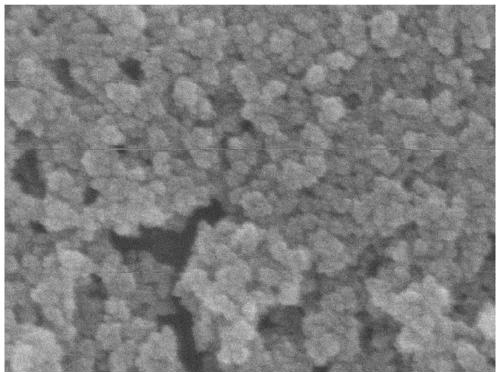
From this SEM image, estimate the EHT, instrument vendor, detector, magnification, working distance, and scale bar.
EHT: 5 kV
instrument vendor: JEOL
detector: SEI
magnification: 160 K X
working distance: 8.5 mm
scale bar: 100 nm